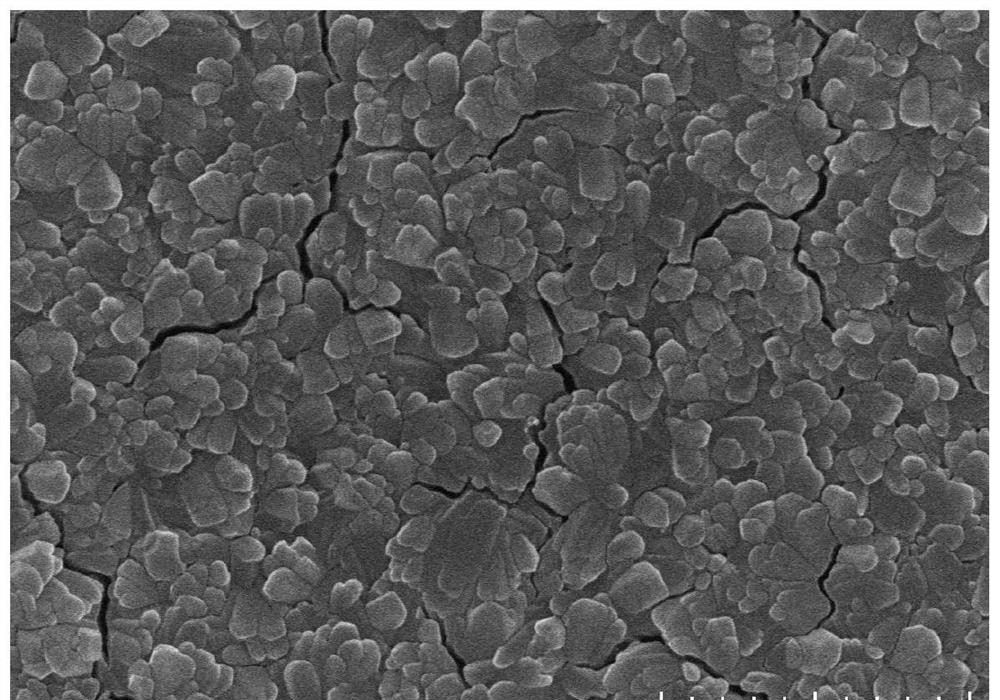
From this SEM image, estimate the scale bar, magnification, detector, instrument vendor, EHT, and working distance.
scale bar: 2000 nm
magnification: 20 K X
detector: SE(U)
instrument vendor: Hitachi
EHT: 10 kV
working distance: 10.6 mm